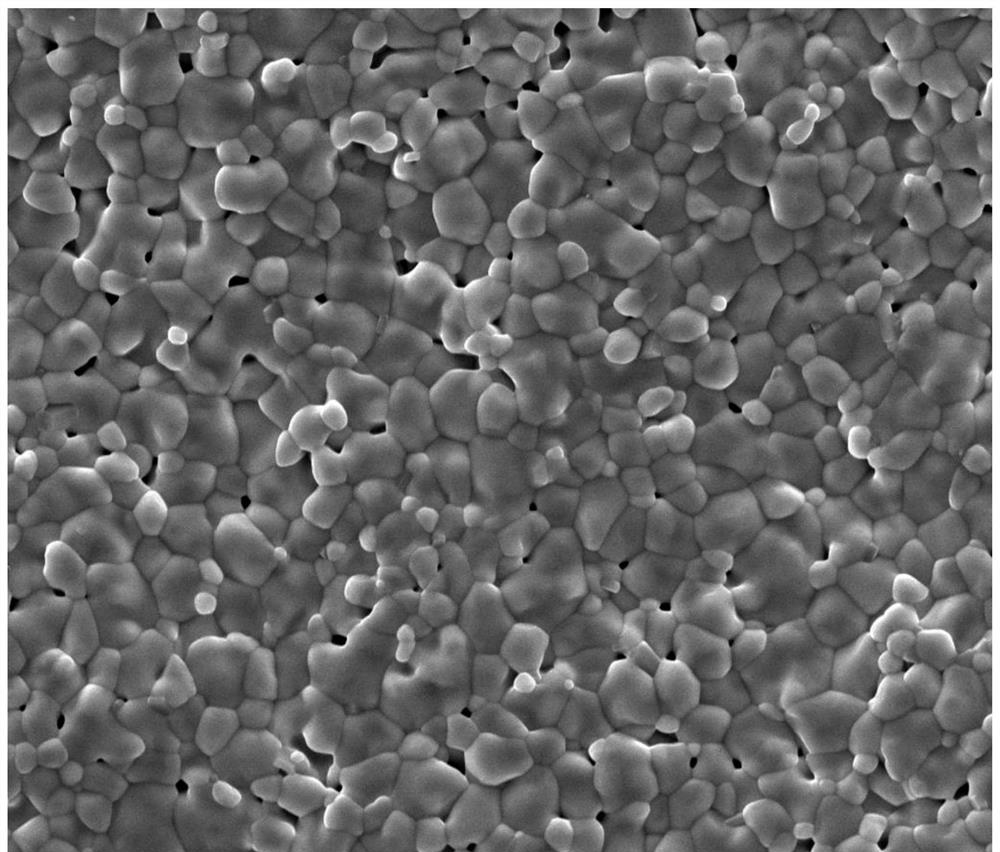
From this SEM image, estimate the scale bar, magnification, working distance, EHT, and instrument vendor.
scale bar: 30000 nm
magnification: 5 K X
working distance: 15.2 mm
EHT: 20 kV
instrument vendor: FEI